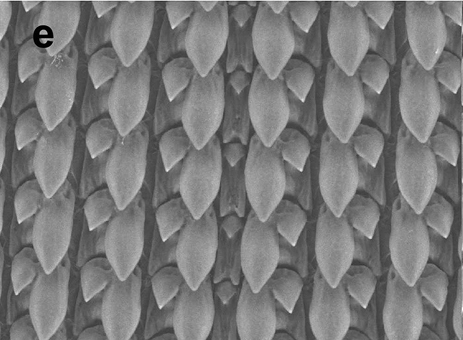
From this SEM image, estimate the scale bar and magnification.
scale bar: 100000 nm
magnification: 0.6 K X